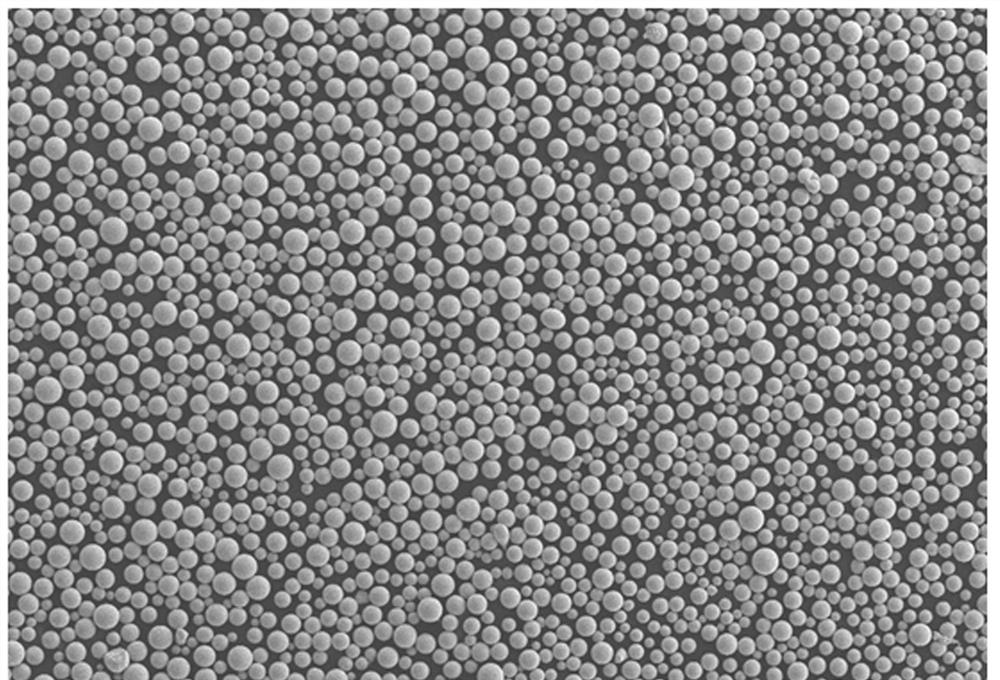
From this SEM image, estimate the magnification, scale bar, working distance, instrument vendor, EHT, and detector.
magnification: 0.05 K X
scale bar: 200000 nm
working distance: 10 mm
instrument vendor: Zeiss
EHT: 15 kV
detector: SE2